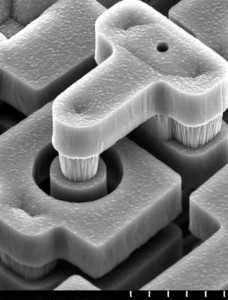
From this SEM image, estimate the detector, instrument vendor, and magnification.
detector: SE(M)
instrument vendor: Hitachi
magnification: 5 K X